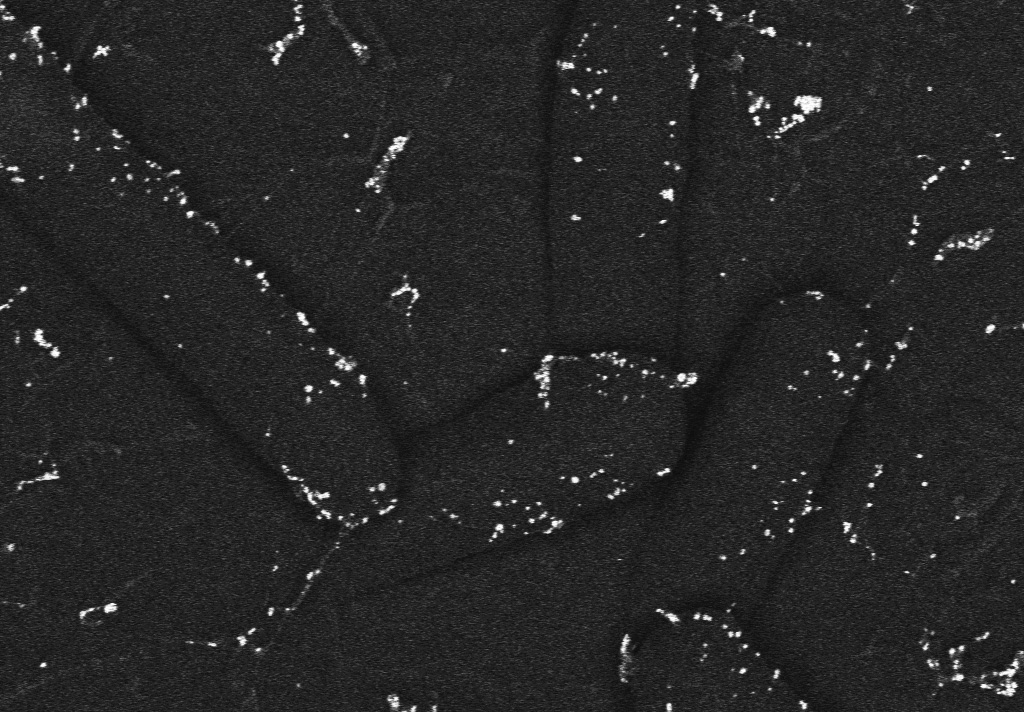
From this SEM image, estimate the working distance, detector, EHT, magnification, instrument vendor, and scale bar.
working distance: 4.2 mm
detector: ESB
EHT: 2 kV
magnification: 20 K X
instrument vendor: Zeiss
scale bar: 200 nm